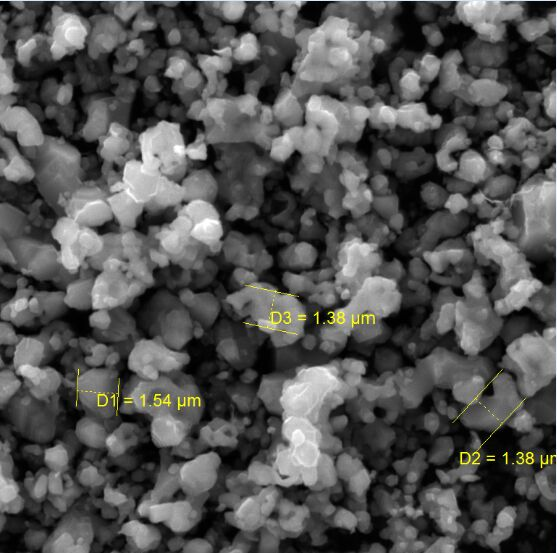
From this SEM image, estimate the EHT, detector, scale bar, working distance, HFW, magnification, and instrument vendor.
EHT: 20 kV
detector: SE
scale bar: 5000 nm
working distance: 10.11 mm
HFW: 20.8 µm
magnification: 10 K X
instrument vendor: Tescan